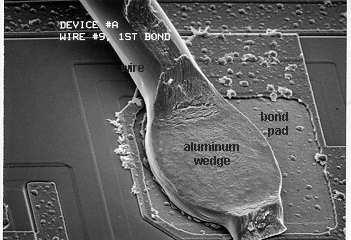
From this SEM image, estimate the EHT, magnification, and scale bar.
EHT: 10 kV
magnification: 0.6 K X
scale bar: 10000 nm